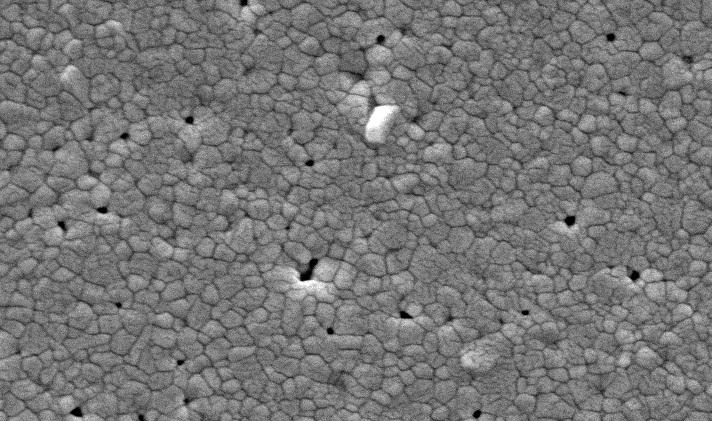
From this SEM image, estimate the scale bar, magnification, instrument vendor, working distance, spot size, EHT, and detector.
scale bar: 2000 nm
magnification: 10 K X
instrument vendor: FEI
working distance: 9.2 mm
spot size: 3.4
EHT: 20 kV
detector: SE